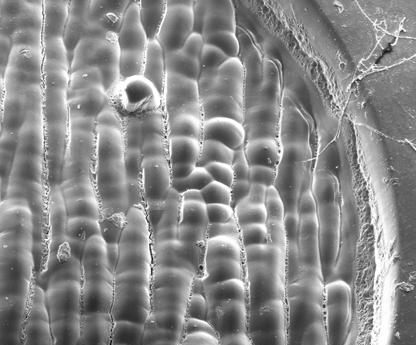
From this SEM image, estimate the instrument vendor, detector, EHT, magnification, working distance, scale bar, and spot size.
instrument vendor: FEI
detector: ETD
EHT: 10 kV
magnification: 0.4 K X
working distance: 8.6 mm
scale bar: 300000 nm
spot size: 3